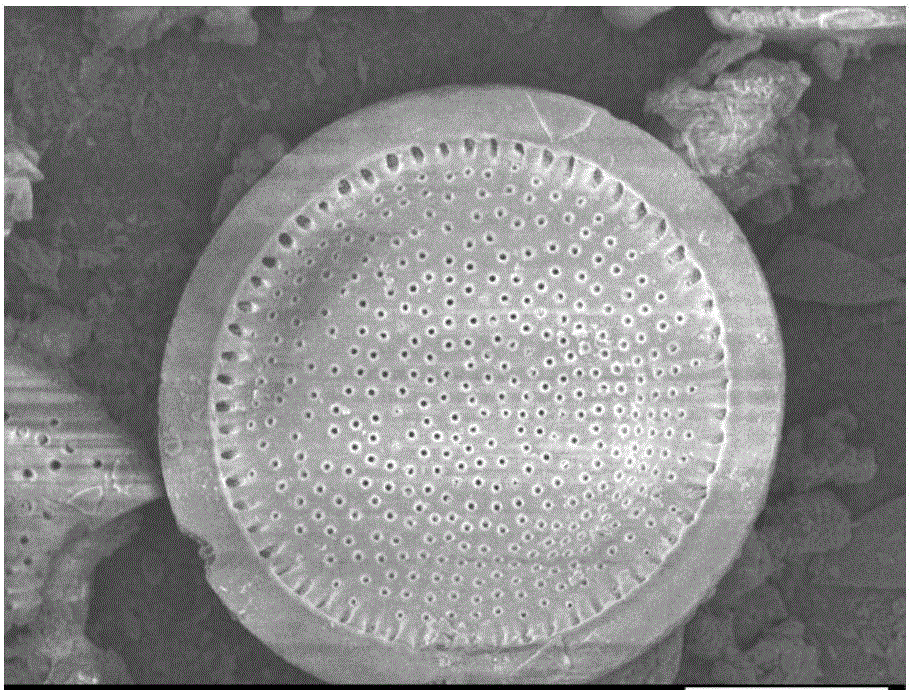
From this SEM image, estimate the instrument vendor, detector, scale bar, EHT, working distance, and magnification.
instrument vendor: JEOL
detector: SEI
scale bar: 10000 nm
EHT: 5 kV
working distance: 6.8 mm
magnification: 2.7 K X